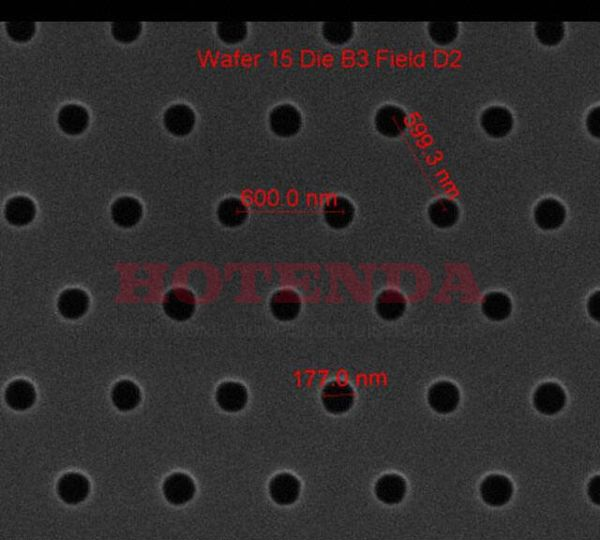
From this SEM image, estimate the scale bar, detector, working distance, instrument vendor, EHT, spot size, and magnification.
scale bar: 1000 nm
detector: ETD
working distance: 10.3 mm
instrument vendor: FEI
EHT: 10 kV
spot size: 1.5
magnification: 75 K X